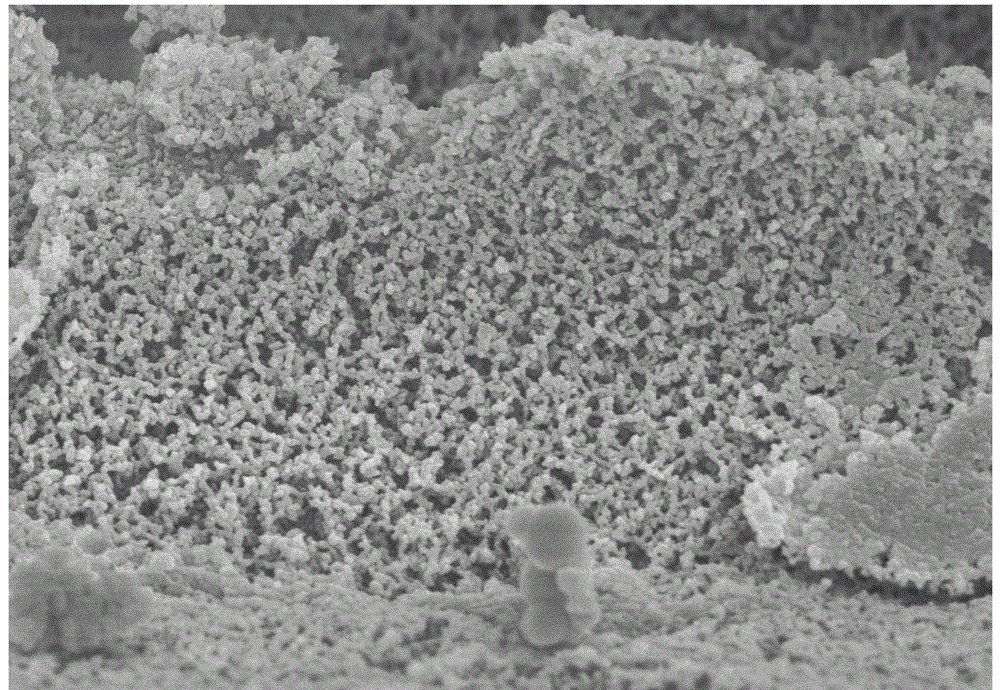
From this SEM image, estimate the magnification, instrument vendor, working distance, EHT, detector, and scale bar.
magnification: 20 K X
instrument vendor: Hitachi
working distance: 8.3 mm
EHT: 3 kV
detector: SE(M)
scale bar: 2000 nm